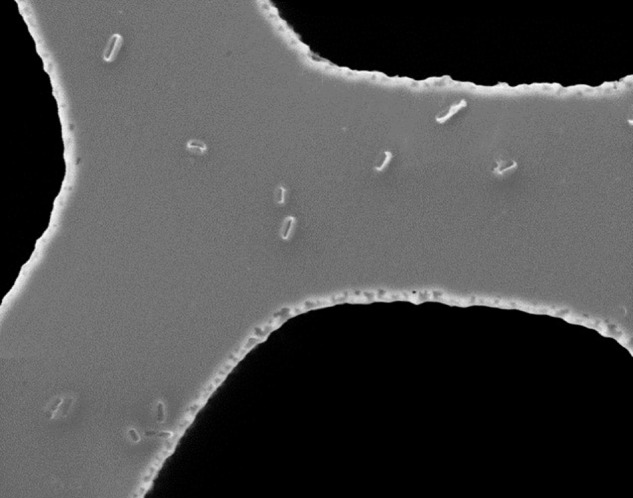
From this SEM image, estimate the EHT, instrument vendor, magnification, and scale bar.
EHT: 10 kV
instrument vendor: JEOL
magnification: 2.2 K X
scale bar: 10000 nm